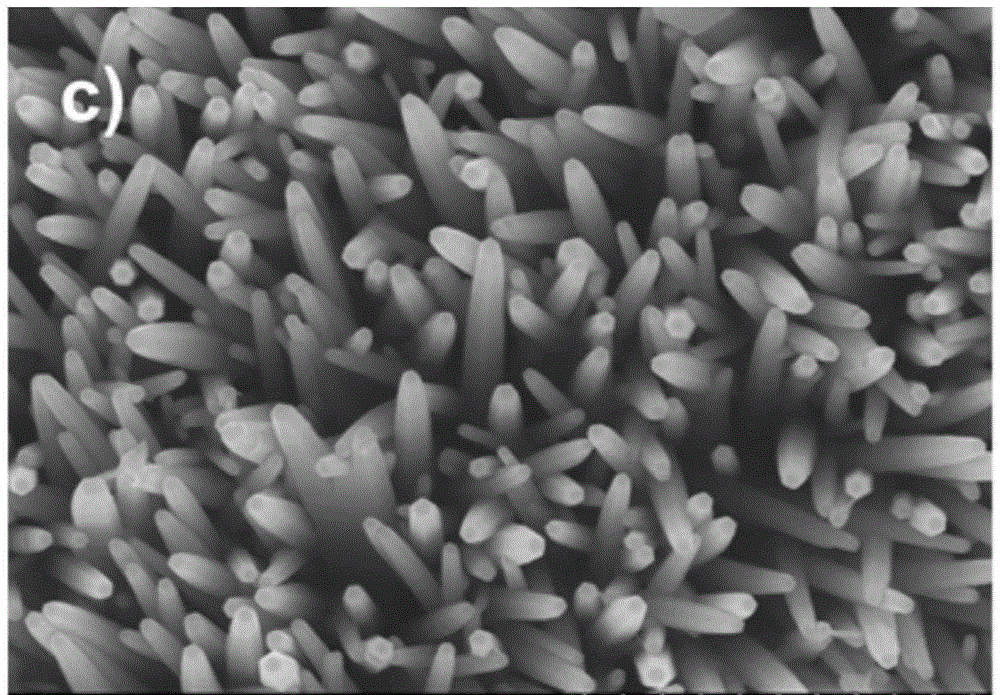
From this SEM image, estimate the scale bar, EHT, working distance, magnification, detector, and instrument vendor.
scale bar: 1000 nm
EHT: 10 kV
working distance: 9.7 mm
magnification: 50 K X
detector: SE(M)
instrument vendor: Hitachi